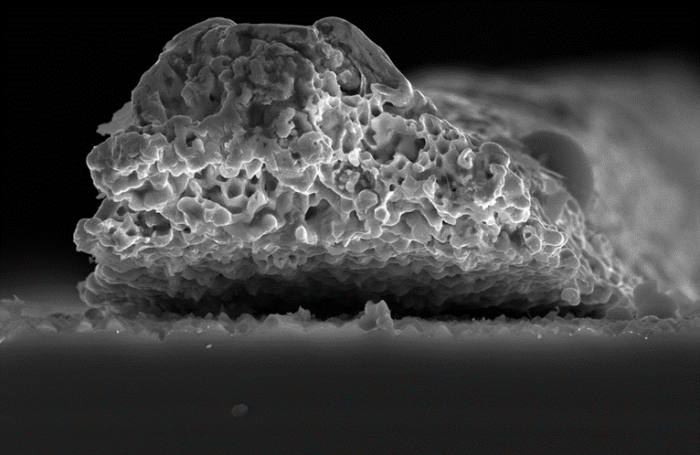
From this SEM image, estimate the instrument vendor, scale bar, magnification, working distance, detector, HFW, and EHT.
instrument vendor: FEI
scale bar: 10000 nm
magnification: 8 K X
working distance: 5.1 mm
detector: ETD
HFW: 51.8 µm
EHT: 20 kV